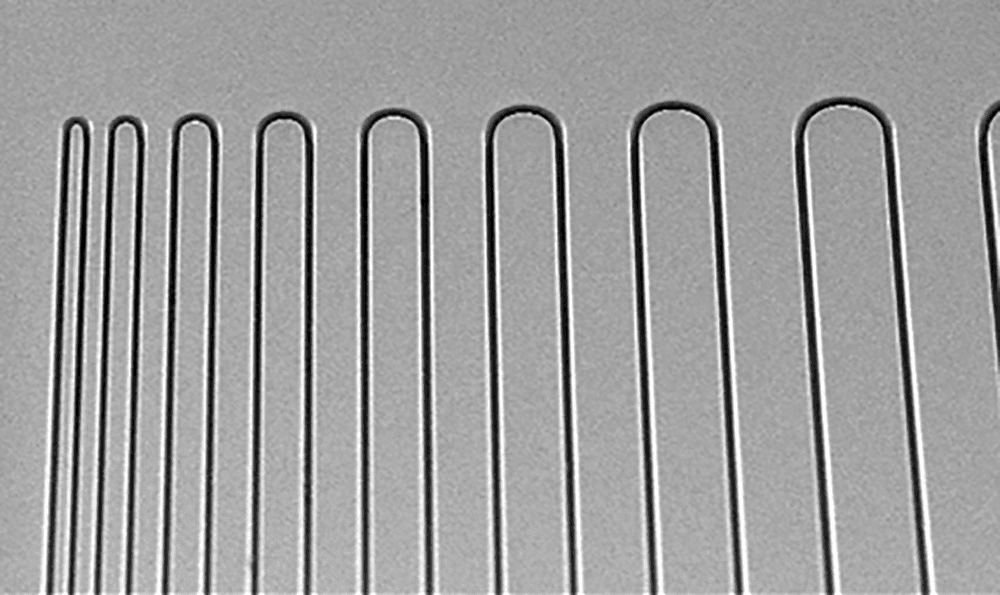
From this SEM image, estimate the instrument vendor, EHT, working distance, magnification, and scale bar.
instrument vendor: JEOL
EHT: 15 kV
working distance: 9.8 mm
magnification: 0.06 K X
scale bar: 500000 nm